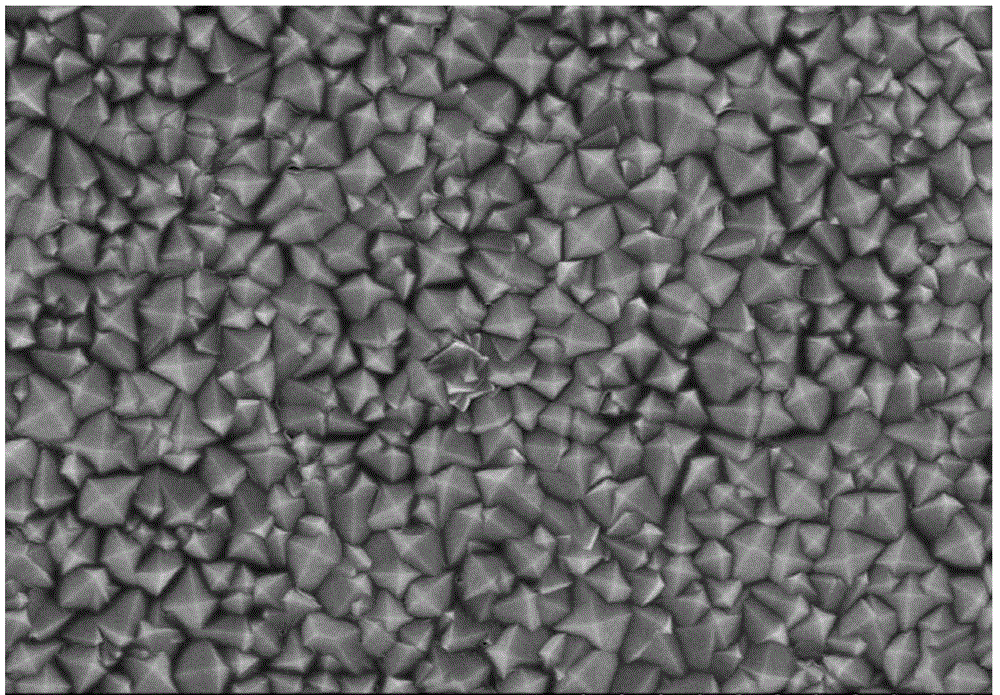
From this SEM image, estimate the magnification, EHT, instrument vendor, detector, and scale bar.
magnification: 10 K X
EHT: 8 kV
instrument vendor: Hitachi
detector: SE(U)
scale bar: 5000 nm